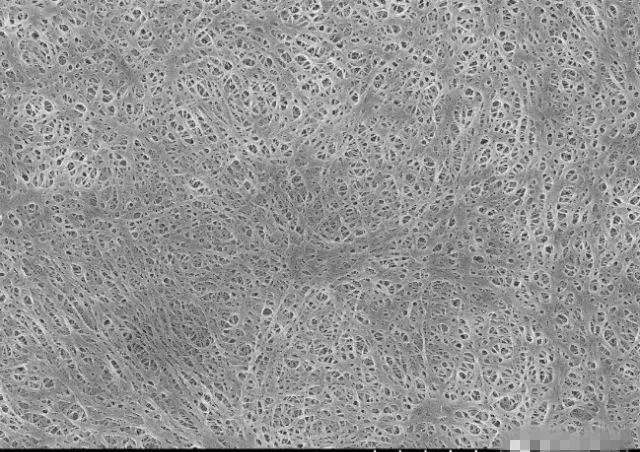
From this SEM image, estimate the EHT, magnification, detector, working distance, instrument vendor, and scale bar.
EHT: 5 kV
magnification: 10 K X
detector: SE(M)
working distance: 8.8 mm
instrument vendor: Hitachi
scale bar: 5000 nm